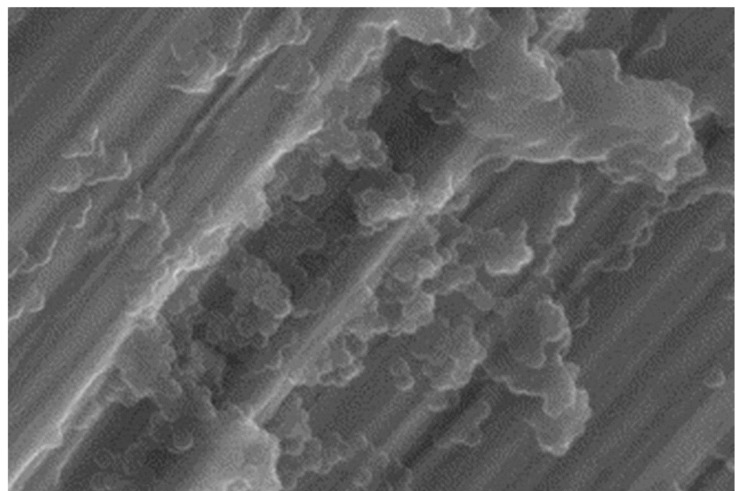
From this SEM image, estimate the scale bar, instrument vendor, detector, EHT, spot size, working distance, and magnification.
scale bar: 2000 nm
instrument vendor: FEI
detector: GSE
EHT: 15 kV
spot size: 4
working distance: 7.9 mm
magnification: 9.911 K X